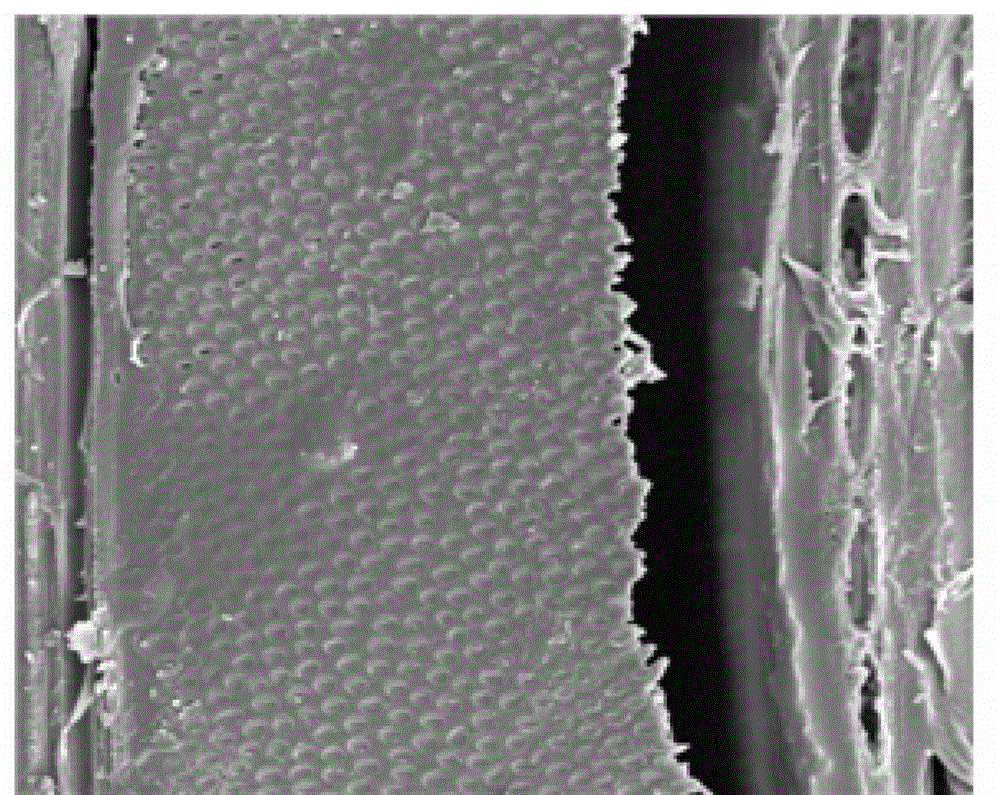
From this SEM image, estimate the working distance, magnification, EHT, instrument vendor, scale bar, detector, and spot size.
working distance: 9.5 mm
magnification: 1 K X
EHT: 10 kV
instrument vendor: FEI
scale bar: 20000 nm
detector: ETD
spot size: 5.5